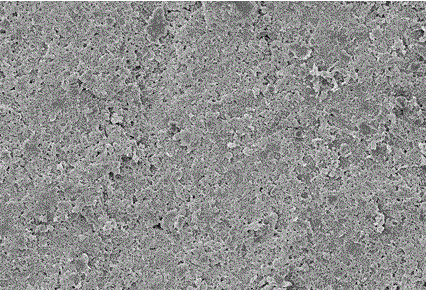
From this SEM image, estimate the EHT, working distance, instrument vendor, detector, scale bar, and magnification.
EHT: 5 kV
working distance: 6.2 mm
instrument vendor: Zeiss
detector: InLens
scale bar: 10000 nm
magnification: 1 K X